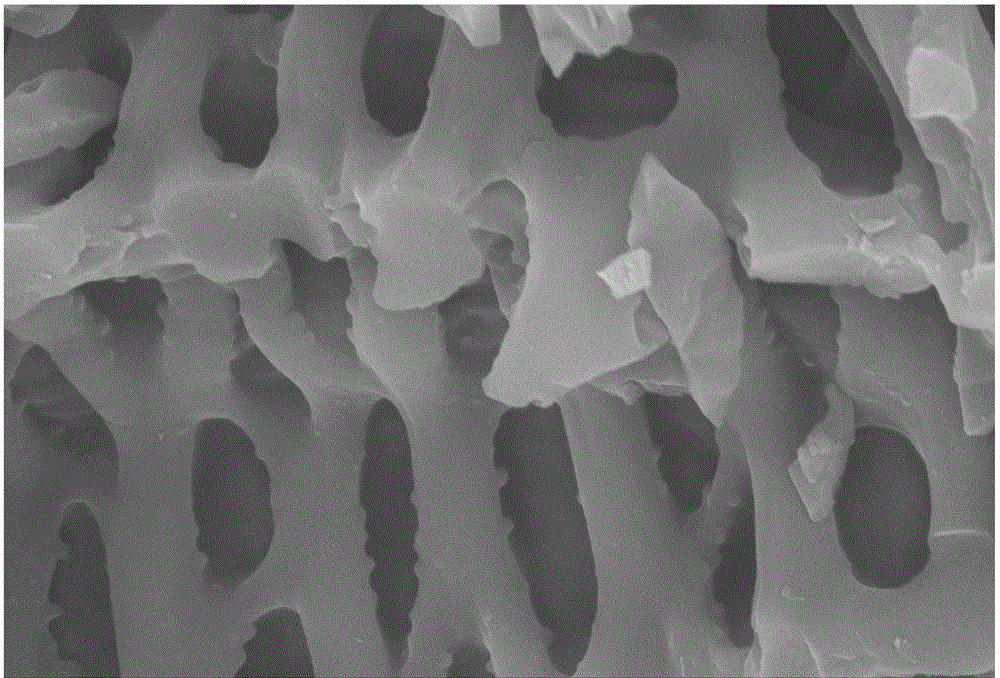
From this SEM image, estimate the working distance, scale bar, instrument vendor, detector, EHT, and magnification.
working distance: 9.6 mm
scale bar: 1000 nm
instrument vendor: Zeiss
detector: SE2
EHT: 10 kV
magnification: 10 K X